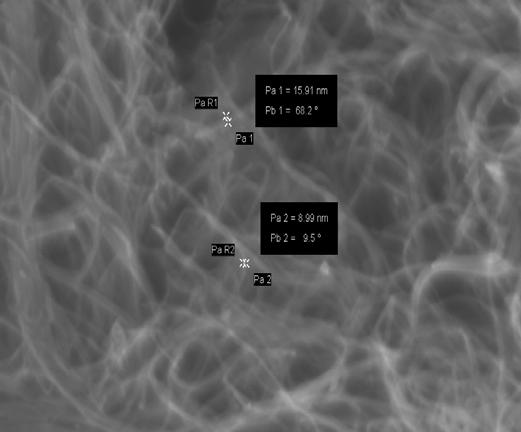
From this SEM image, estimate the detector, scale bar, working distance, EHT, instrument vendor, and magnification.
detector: InLens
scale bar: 200 nm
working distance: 4 mm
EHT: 10 kV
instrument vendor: Zeiss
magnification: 75.57 K X